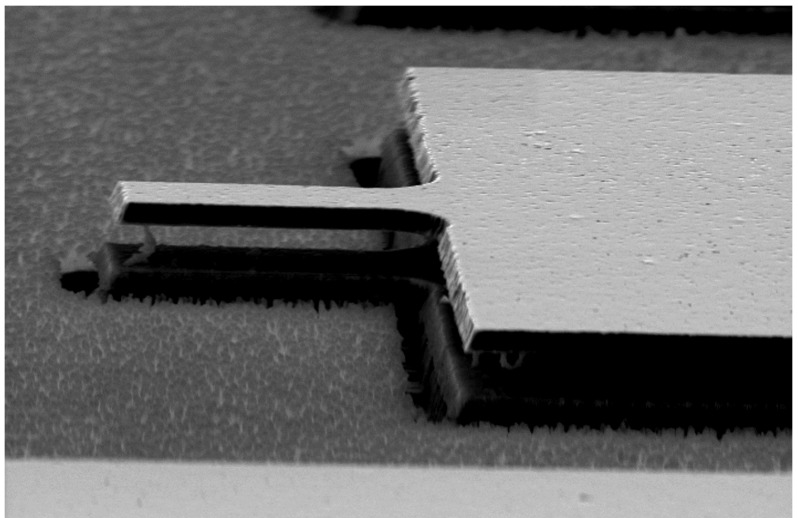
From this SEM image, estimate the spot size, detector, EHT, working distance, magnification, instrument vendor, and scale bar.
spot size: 3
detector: SE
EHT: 10 kV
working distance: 15.1 mm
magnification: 2 K X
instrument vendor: FEI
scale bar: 10000 nm